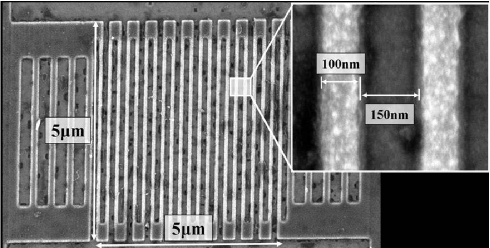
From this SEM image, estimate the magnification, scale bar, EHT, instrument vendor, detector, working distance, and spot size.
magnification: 12 K X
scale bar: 2000 nm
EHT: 3 kV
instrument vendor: FEI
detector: TLD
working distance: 1.7 mm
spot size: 2